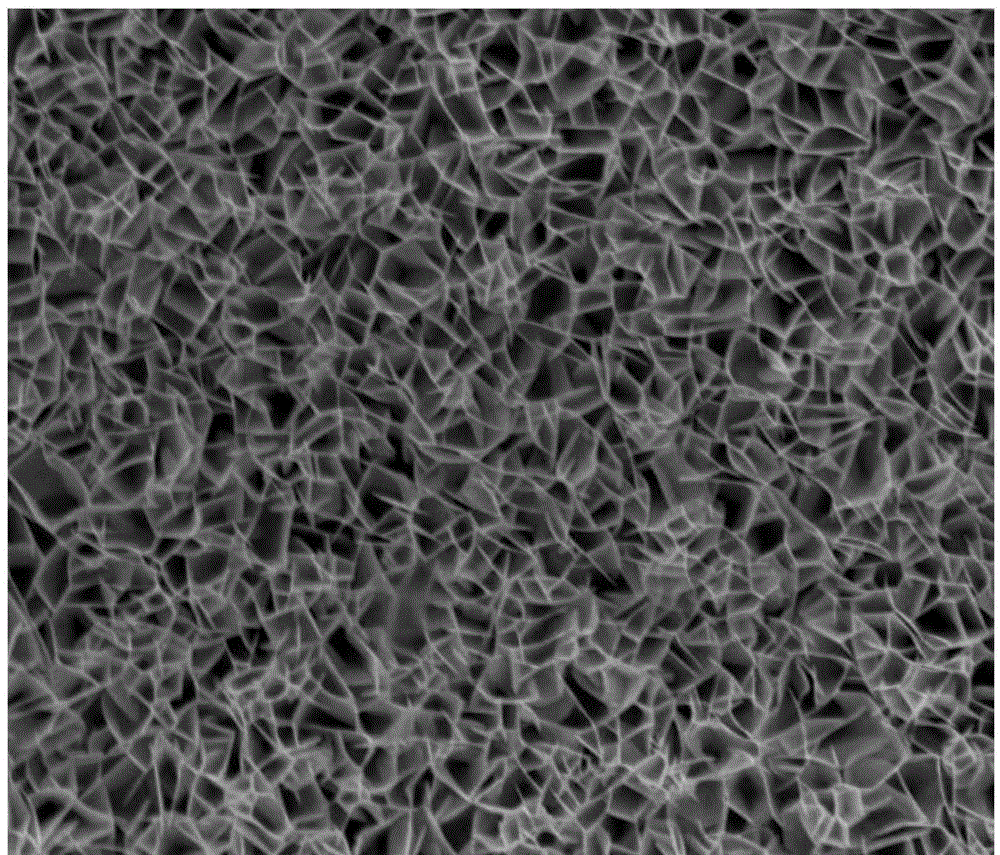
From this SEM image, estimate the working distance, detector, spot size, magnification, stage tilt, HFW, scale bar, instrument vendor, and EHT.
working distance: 12.1 mm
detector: ETD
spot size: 3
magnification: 5 K X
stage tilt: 0°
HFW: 59.7 µm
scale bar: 20000 nm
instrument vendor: FEI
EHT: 20 kV